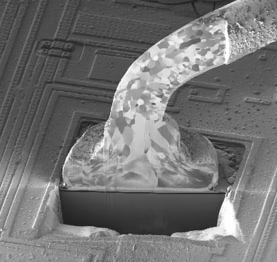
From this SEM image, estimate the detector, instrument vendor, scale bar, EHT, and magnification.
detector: SIM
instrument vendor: Hitachi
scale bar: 30000 nm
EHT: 30 kV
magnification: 1.5 K X